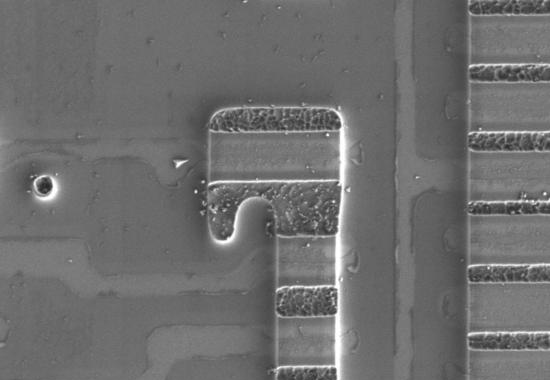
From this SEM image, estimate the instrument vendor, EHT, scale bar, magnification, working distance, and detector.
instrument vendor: Hitachi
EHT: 1.5 kV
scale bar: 5000 nm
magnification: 11 K X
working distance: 8.7 mm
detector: SE(L)